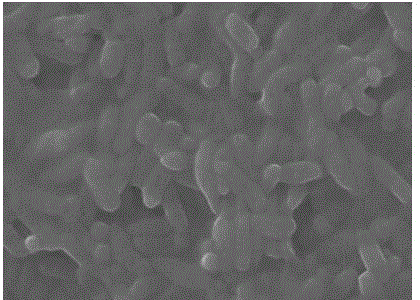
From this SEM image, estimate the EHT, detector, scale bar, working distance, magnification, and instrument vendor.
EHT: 10 kV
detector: LED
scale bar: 1000 nm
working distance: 9.7 mm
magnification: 14 K X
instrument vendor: JEOL